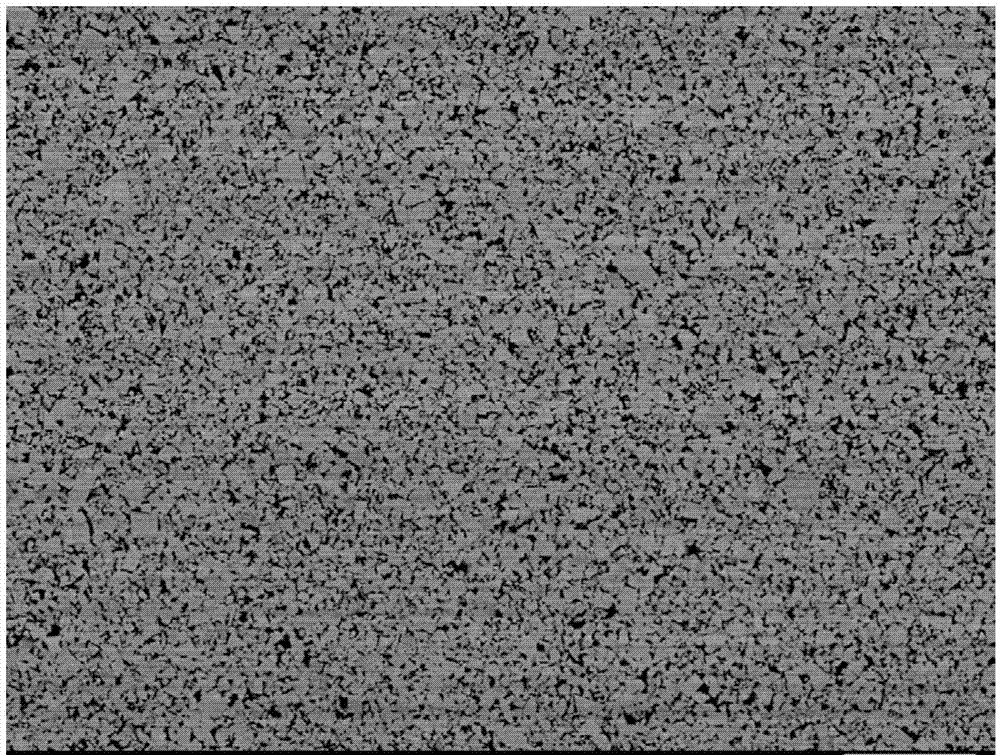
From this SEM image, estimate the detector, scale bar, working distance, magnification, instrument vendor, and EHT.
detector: COMPO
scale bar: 10000 nm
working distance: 8.3 mm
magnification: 1.6 K X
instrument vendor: JEOL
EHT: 15 kV